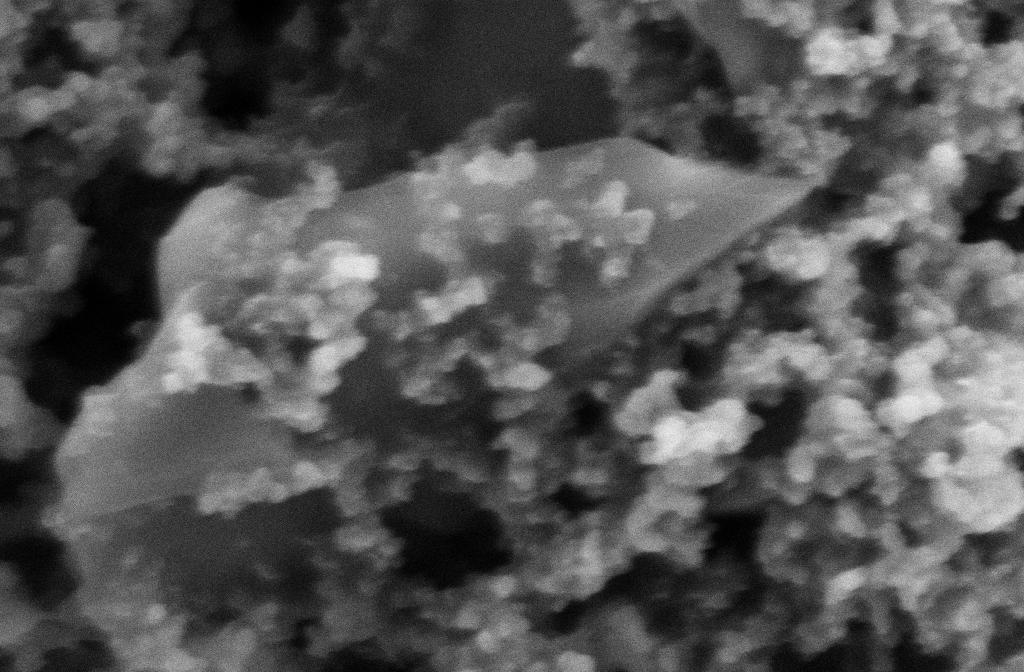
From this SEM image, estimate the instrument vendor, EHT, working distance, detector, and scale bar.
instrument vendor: Zeiss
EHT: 5 kV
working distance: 8 mm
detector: SE2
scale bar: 200 nm